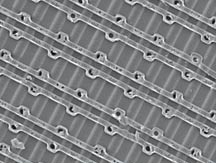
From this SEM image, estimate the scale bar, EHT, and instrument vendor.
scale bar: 10000 nm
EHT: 5 kV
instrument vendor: JEOL